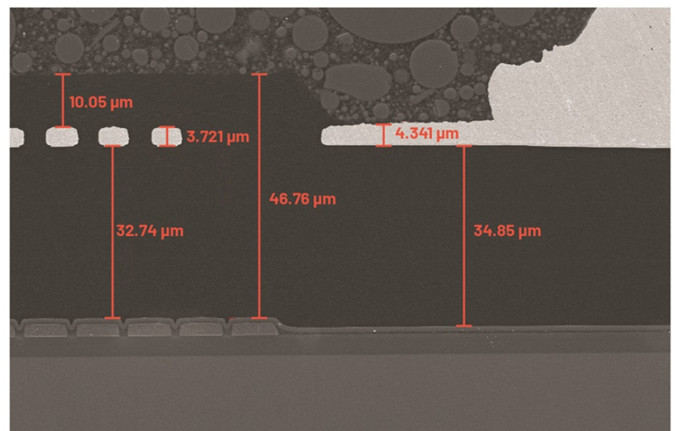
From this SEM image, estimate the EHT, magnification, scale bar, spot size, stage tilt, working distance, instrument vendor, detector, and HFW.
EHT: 10 kV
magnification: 1 K X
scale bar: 50000 nm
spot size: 3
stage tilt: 0°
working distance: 10 mm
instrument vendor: FEI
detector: ETD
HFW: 127 µm